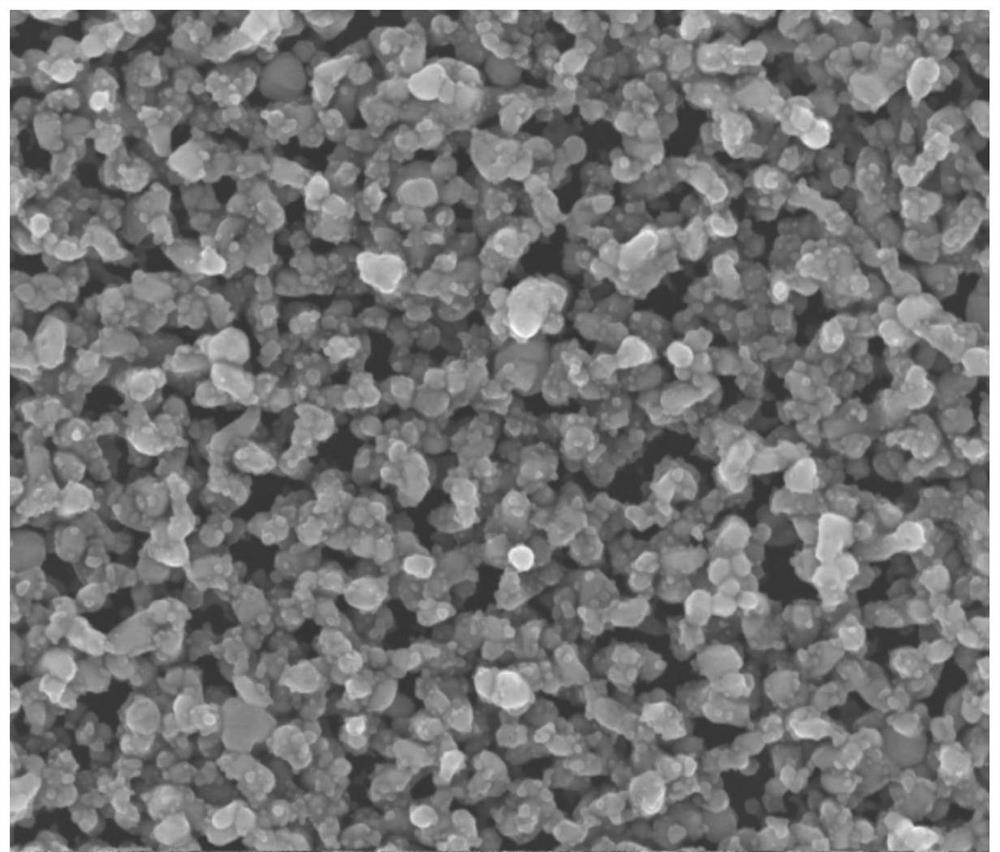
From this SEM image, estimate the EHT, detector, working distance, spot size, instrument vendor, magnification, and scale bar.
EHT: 15 kV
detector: TLD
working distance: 5.7 mm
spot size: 3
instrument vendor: FEI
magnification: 50 K X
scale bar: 1000 nm